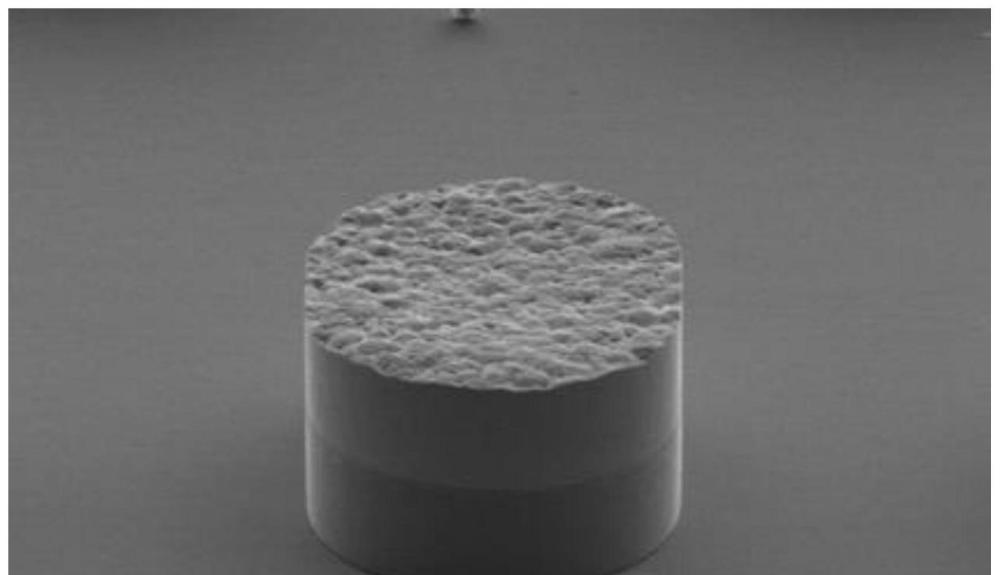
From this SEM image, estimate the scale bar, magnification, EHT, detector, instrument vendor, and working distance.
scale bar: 100000 nm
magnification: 0.5 K X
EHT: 15 kV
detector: SE(M)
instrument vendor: Hitachi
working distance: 9.1 mm